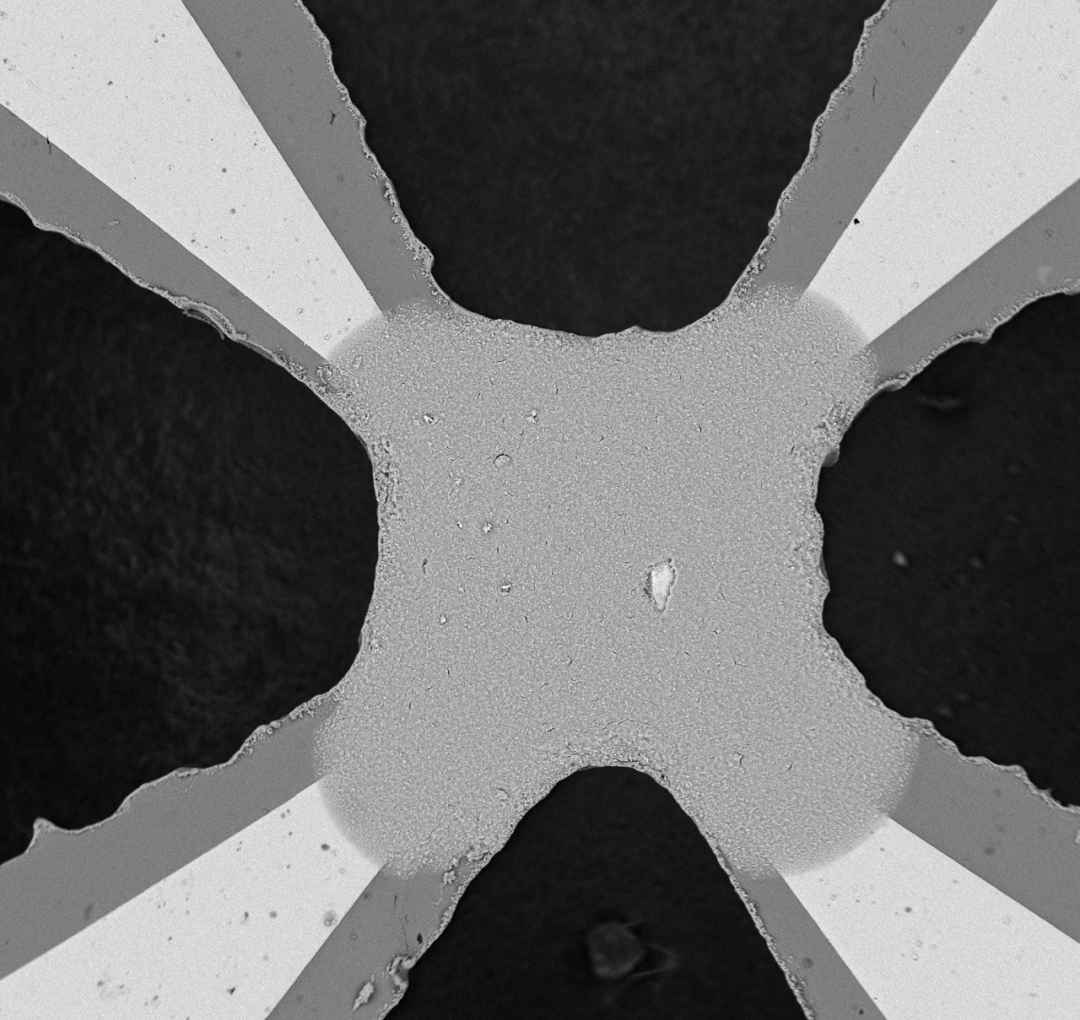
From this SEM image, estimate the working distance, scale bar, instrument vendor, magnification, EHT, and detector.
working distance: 7.1 mm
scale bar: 100000 nm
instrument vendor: other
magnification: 0.5 K X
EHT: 15 kV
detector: Mix 50%